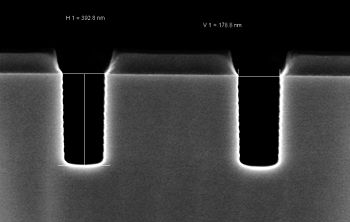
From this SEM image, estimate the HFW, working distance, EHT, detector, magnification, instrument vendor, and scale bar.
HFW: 1.501 µm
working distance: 3.2 mm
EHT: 2 kV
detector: InLens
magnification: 200 K X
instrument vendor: Zeiss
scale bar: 100 nm